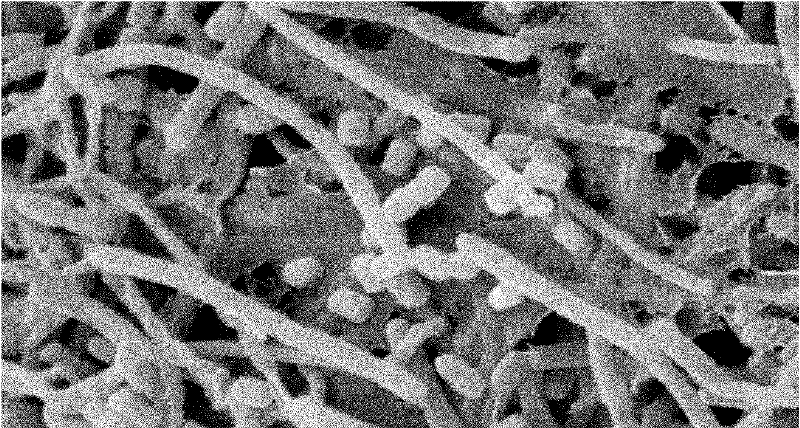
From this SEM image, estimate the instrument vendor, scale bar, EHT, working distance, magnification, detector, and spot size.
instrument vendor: FEI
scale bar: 2000 nm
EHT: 10 kV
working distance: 7.9 mm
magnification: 8 K X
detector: SE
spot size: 3